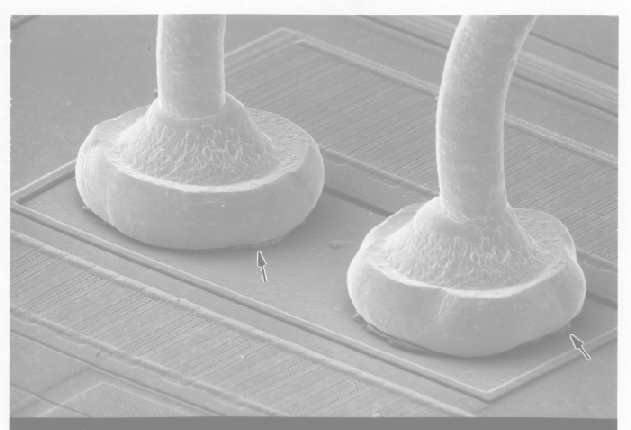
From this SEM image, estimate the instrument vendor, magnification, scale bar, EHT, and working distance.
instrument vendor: JEOL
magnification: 0.5 K X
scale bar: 10000 nm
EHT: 15 kV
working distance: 48 mm